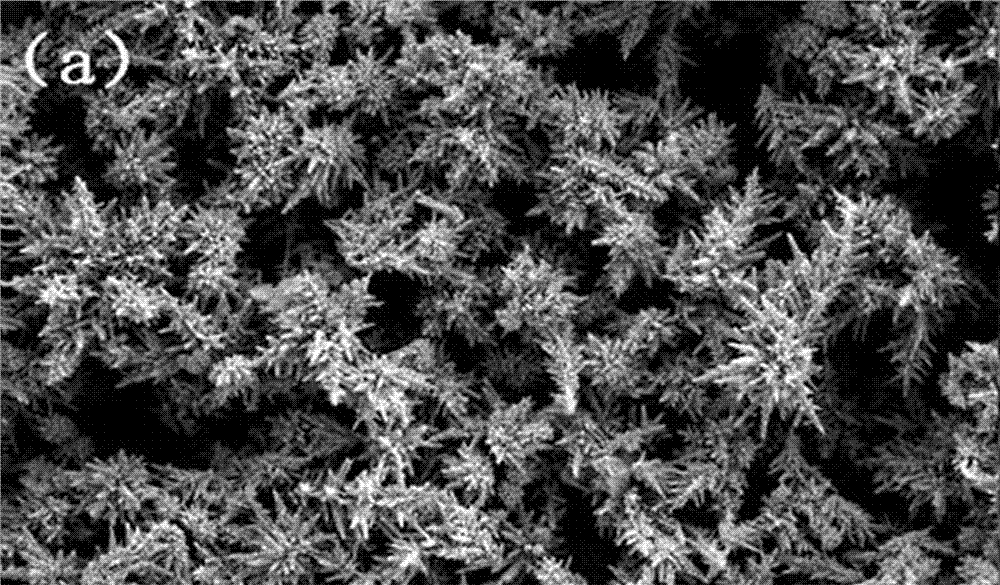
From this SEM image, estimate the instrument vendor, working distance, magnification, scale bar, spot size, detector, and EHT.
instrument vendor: FEI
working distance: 7.3 mm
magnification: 5 K X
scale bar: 10000 nm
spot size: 3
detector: SE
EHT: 5 kV